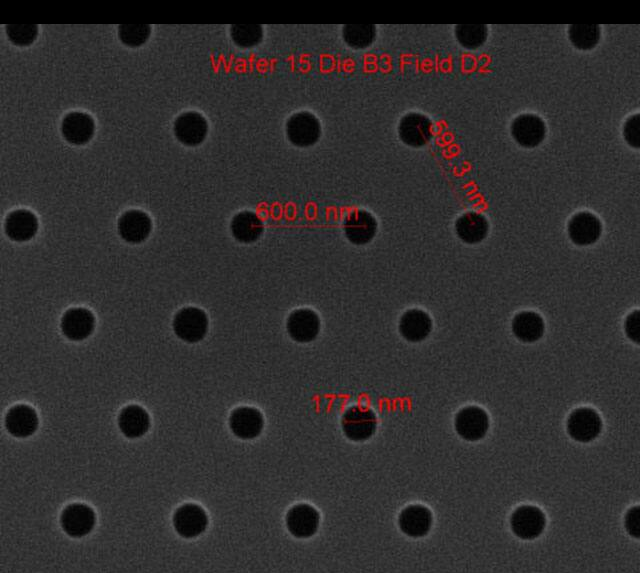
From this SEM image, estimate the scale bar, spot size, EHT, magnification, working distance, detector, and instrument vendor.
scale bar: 1000 nm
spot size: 1.5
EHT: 10 kV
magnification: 75 K X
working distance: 10.3 mm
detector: ETD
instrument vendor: FEI